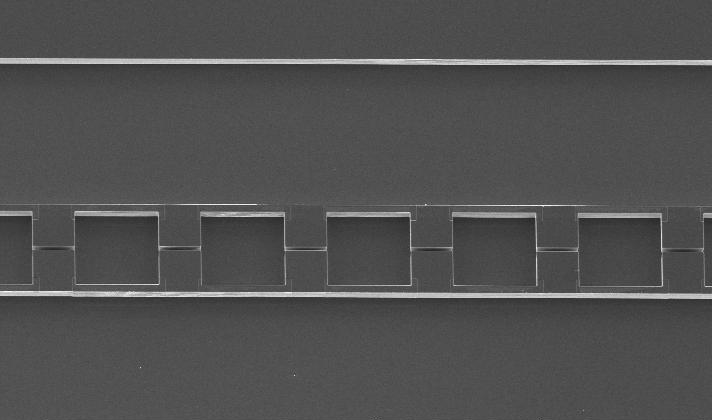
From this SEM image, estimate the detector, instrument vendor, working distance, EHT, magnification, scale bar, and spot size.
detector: SE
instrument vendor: FEI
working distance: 18.1 mm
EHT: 10 kV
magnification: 0.147 K X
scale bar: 200000 nm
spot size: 3